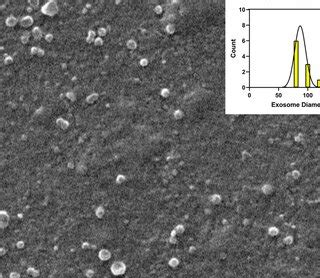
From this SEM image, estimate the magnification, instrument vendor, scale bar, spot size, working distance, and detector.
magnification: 10 K X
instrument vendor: FEI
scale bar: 2000 nm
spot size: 2.2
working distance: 15.3 mm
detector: SE